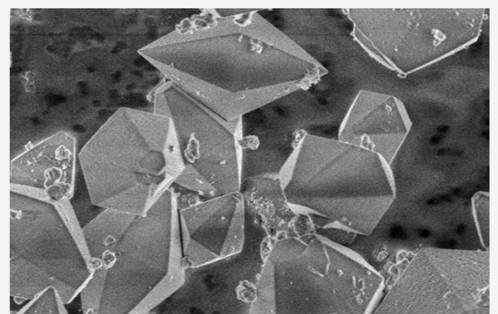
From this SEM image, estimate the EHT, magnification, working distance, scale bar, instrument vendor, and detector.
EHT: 2 kV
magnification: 5 K X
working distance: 9 mm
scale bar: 10000 nm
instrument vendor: Hitachi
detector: SE(M)